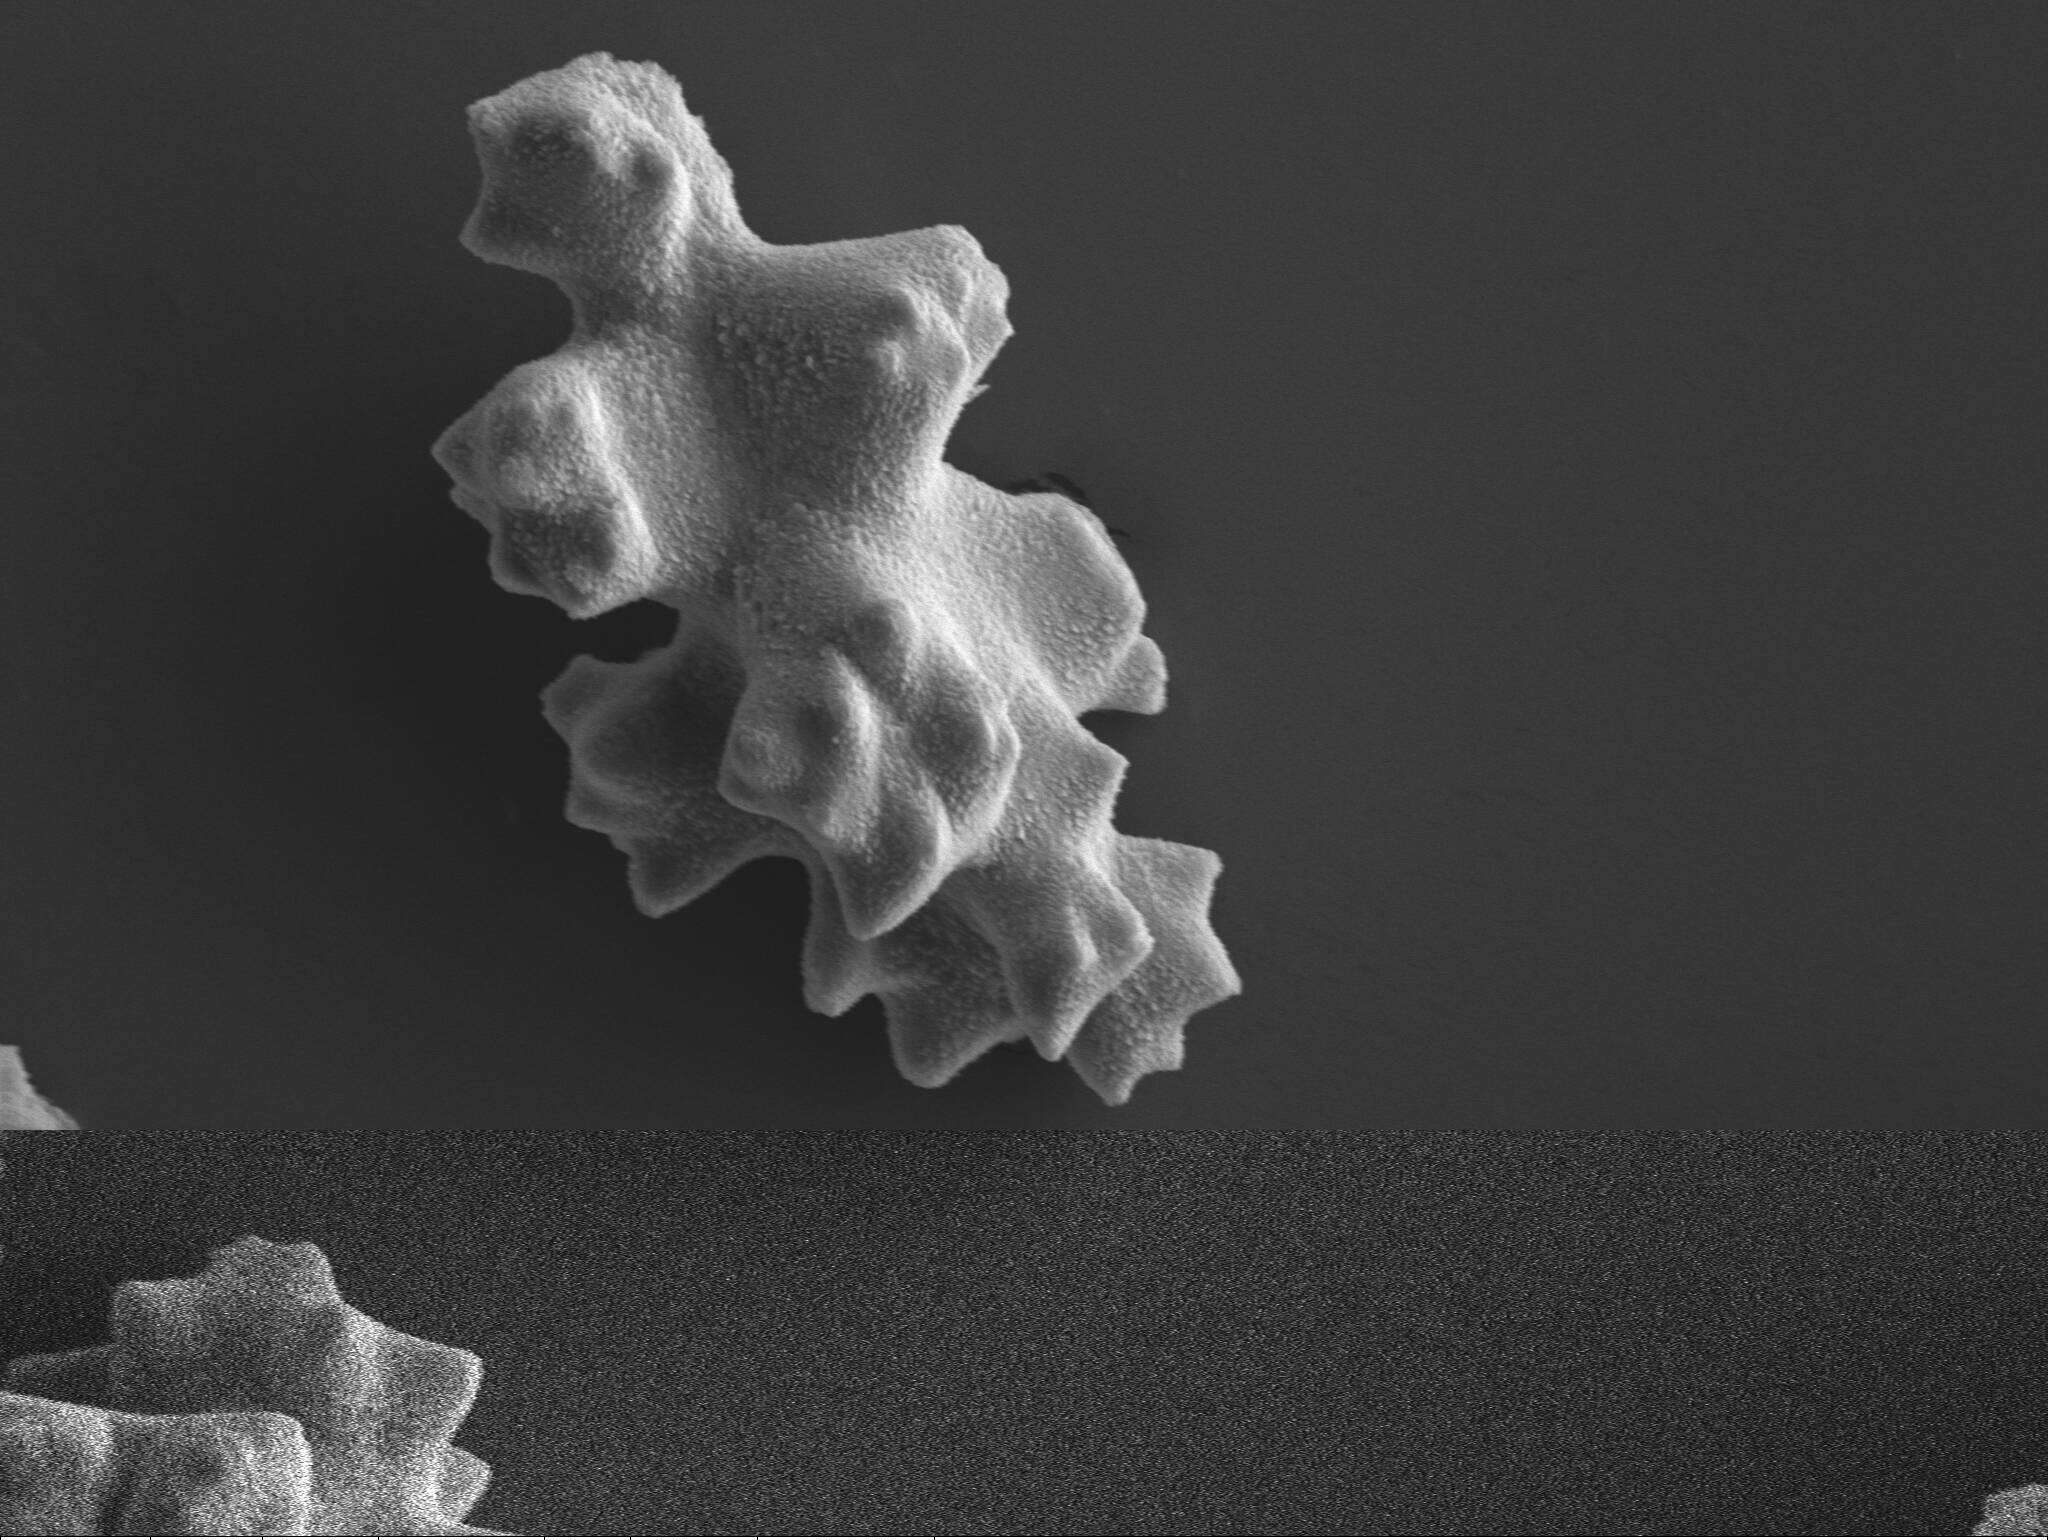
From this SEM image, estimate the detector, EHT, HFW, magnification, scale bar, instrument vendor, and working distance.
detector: SE1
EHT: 12 kV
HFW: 114.4 µm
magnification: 1 K X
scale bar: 20000 nm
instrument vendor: Zeiss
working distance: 13.3 mm